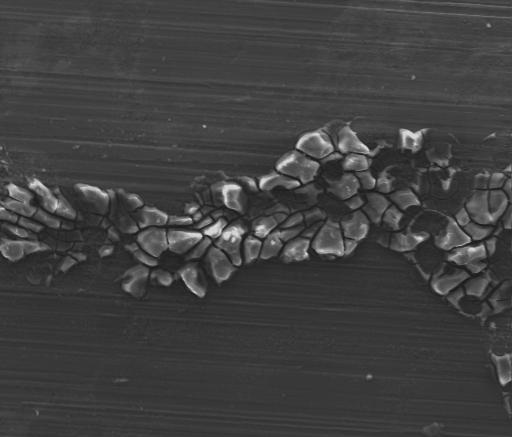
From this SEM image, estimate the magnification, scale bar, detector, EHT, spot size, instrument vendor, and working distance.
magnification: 0.2 K X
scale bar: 300000 nm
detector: Mix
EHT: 25 kV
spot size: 5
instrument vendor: FEI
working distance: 12 mm